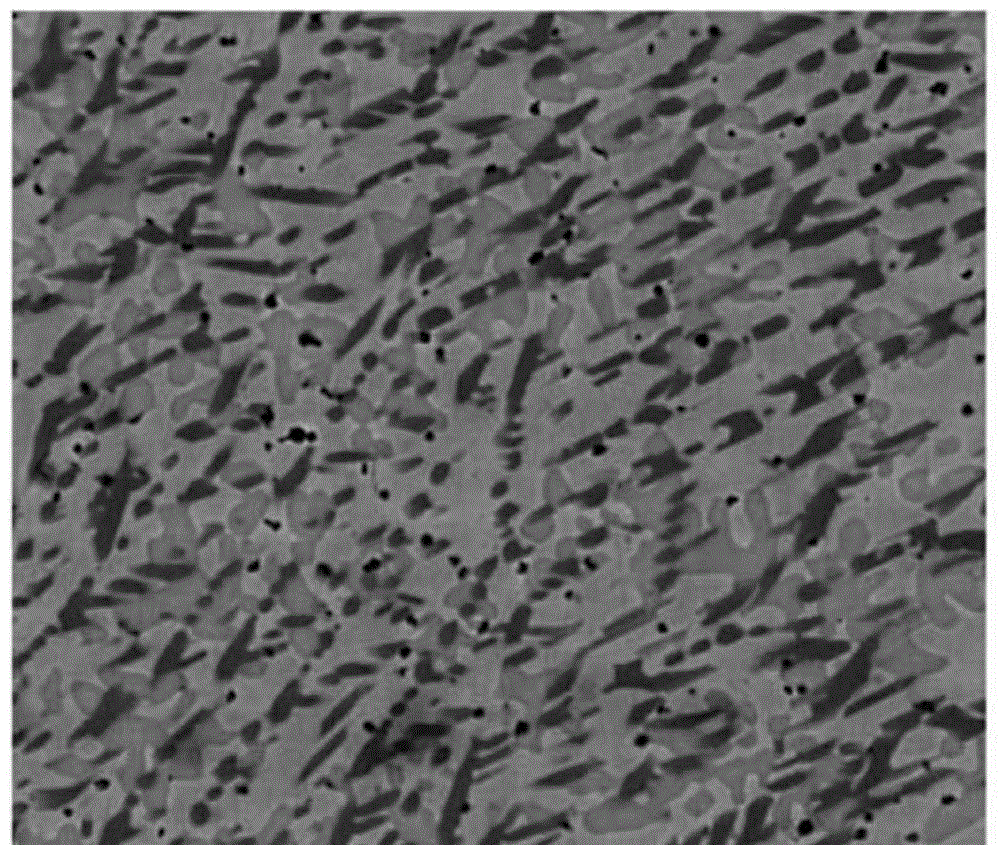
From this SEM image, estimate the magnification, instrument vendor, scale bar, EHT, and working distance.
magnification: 5 K X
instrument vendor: FEI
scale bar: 20000 nm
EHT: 20 kV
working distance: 5.3 mm